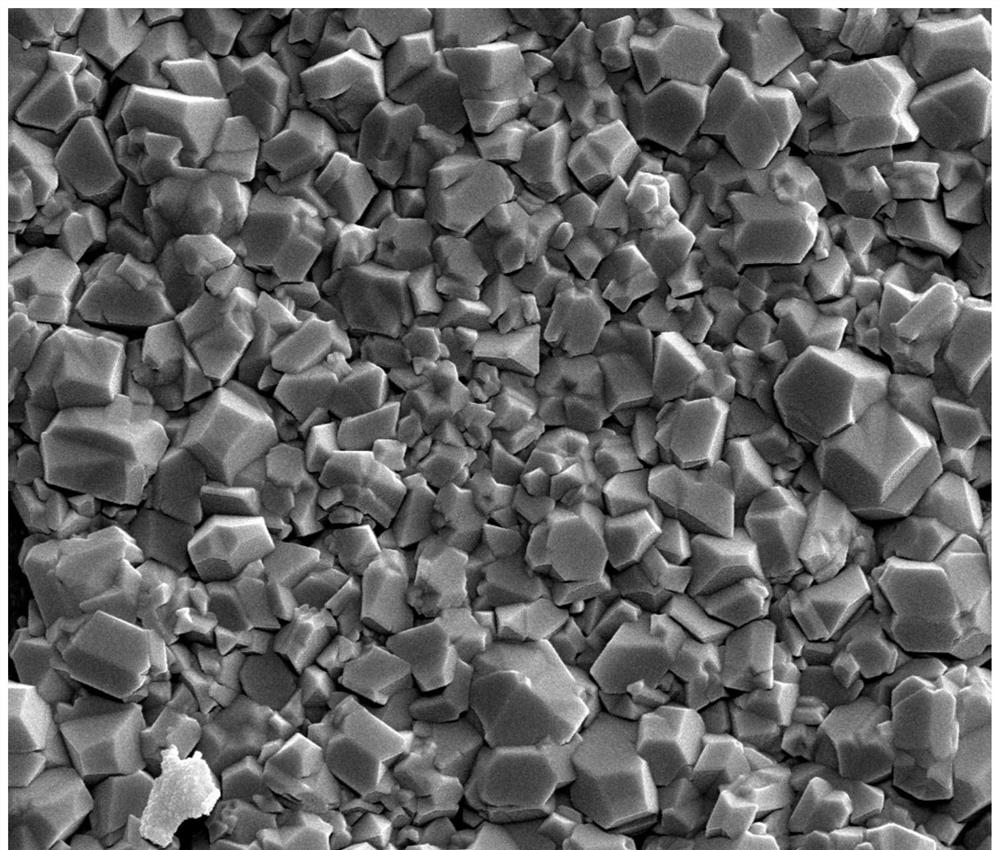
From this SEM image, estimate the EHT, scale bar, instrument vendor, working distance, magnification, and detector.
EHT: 20 kV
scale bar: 4000 nm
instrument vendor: FEI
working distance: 5.2 mm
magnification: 20 K X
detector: SE